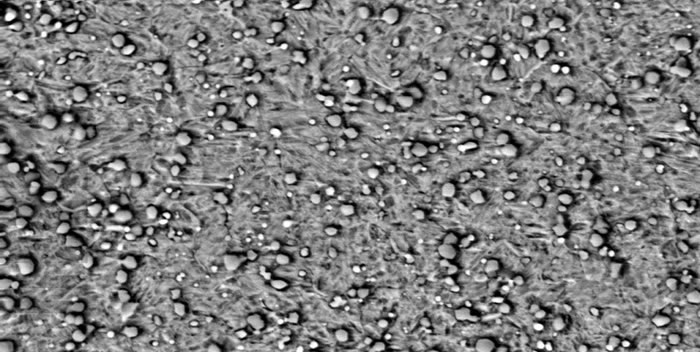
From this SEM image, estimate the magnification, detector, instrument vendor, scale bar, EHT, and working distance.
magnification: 2 K X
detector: CZ BSD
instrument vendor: Zeiss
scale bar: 10000 nm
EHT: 19.99 kV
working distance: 13.2 mm